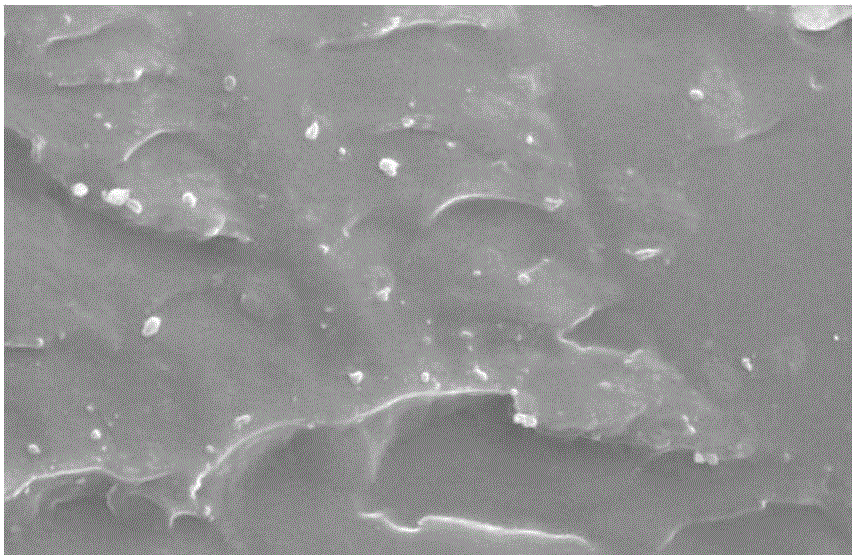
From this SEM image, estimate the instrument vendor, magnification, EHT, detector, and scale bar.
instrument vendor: JEOL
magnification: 2 K X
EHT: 20 kV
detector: SEI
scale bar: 10000 nm